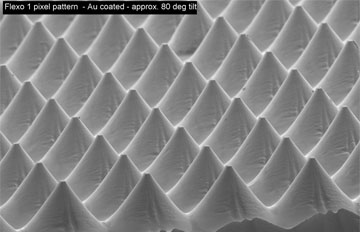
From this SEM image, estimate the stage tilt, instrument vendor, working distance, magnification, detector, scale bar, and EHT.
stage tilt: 80°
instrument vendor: Zeiss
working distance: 15 mm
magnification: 0.2 K X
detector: SE2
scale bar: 100000 nm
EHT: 5 kV